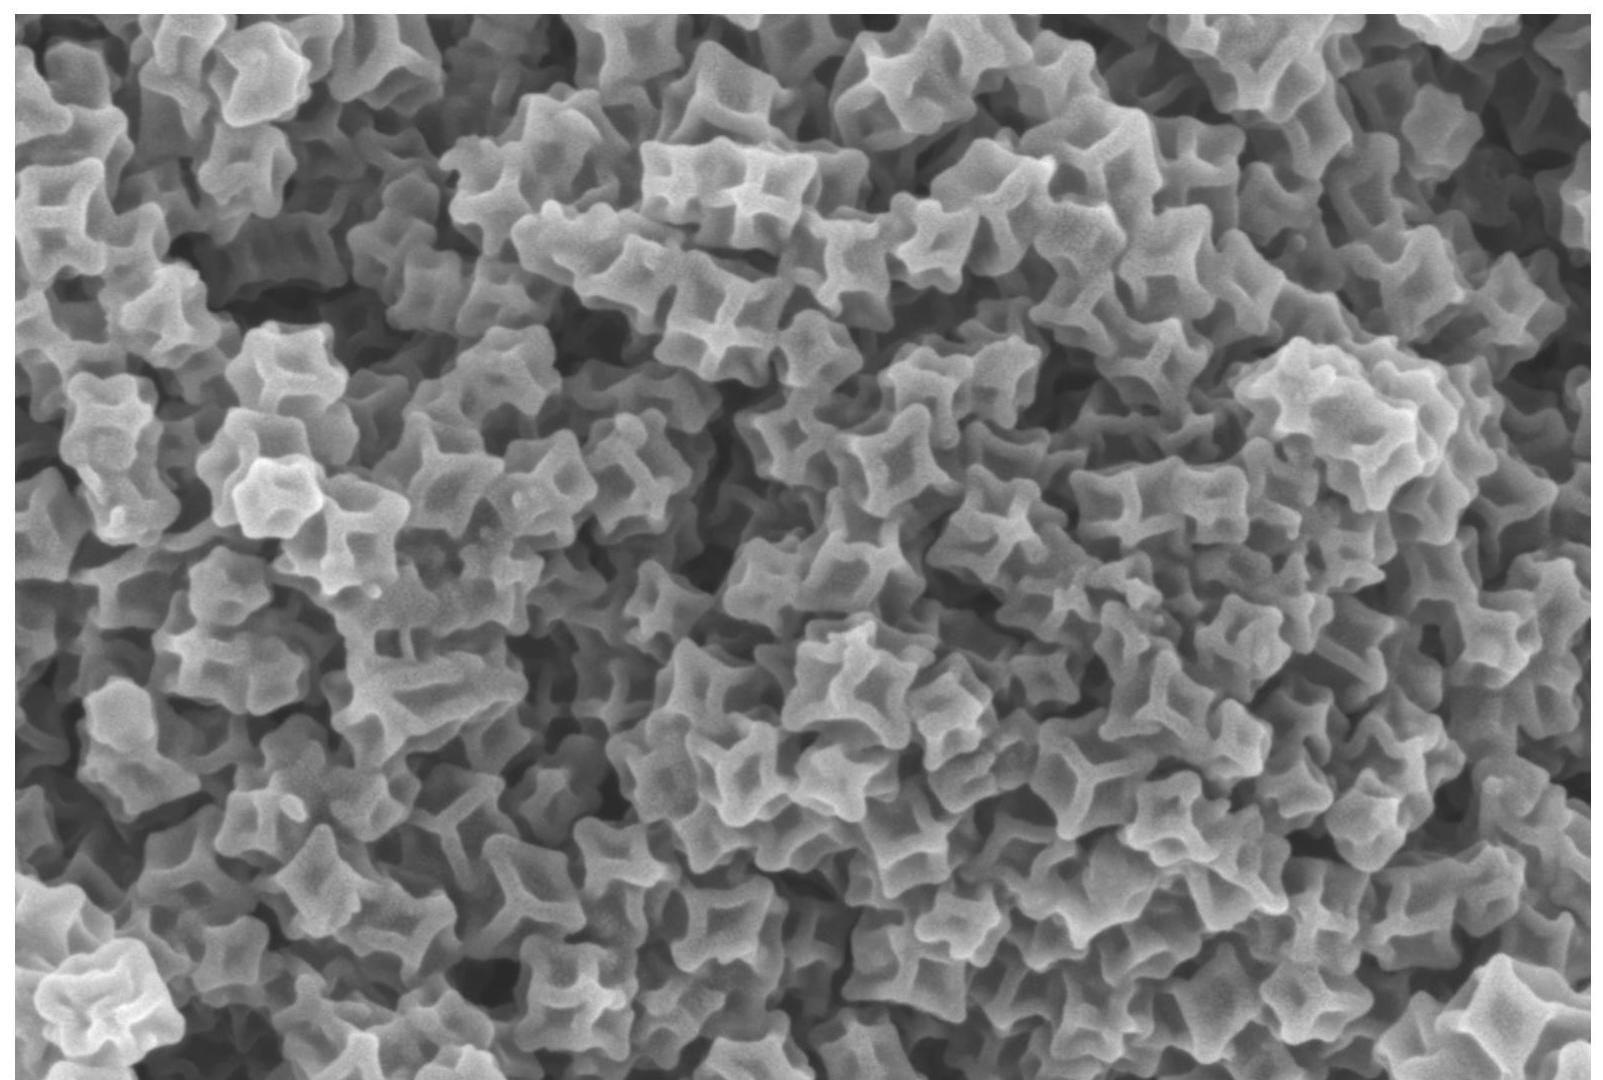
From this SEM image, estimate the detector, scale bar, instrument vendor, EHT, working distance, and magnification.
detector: InLens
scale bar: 200 nm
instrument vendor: Zeiss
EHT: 3 kV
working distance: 6.5 mm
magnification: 50 K X